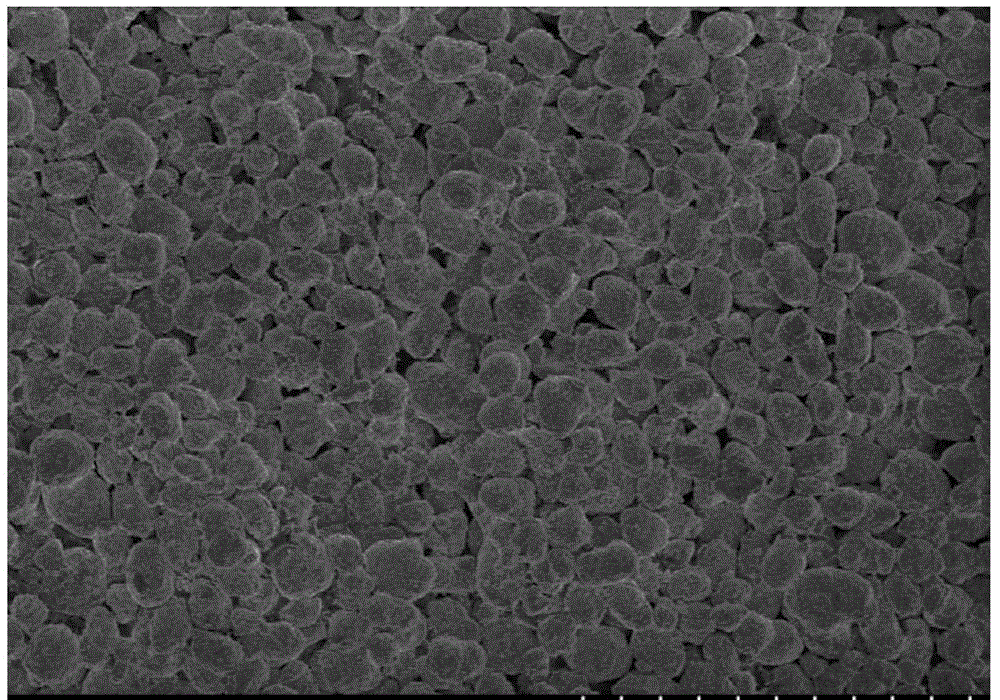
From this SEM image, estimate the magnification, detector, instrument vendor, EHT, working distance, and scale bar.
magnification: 1 K X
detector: SE(M)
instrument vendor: Hitachi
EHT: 3 kV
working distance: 10 mm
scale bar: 50000 nm